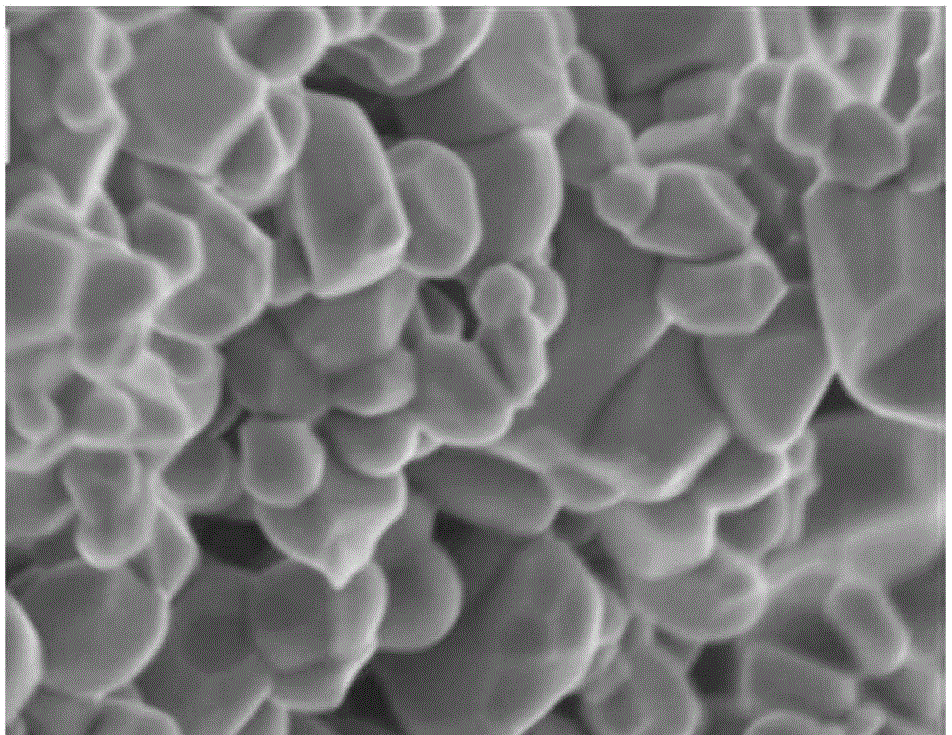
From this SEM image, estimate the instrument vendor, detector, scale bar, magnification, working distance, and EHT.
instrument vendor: Hitachi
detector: SE+BSE(TU)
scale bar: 500 nm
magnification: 100 K X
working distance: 6.6 mm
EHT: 2 kV